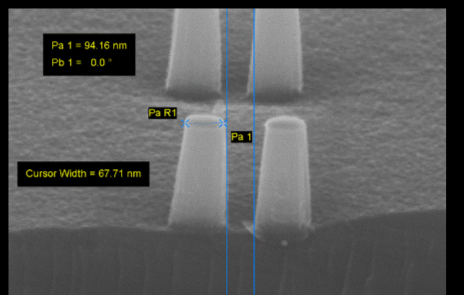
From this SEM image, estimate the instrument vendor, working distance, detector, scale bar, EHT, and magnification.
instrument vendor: Zeiss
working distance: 7 mm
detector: InLens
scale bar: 100 nm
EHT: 20 kV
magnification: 105.53 K X